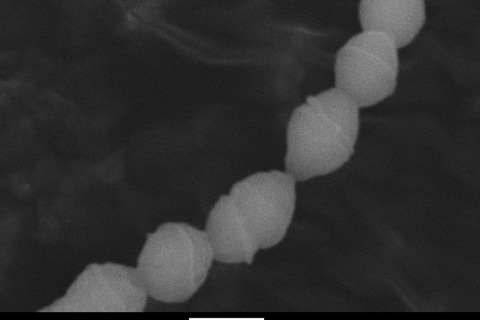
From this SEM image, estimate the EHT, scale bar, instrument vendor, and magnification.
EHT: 10 kV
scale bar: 1000 nm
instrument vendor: JEOL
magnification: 20 K X